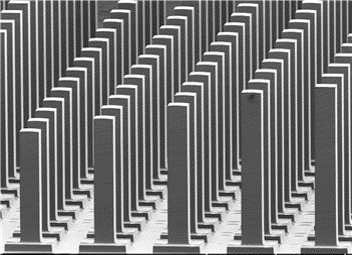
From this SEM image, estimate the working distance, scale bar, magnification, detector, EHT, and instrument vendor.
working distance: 16.3 mm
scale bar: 1000000 nm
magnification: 0.05 K X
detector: LM(UL)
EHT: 3 kV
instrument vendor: Hitachi